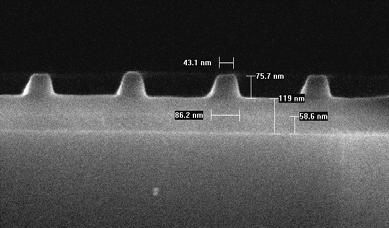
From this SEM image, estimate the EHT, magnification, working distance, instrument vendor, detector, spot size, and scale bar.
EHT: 10 kV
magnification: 100 K X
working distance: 5.1 mm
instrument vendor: FEI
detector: TLD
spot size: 2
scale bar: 200 nm